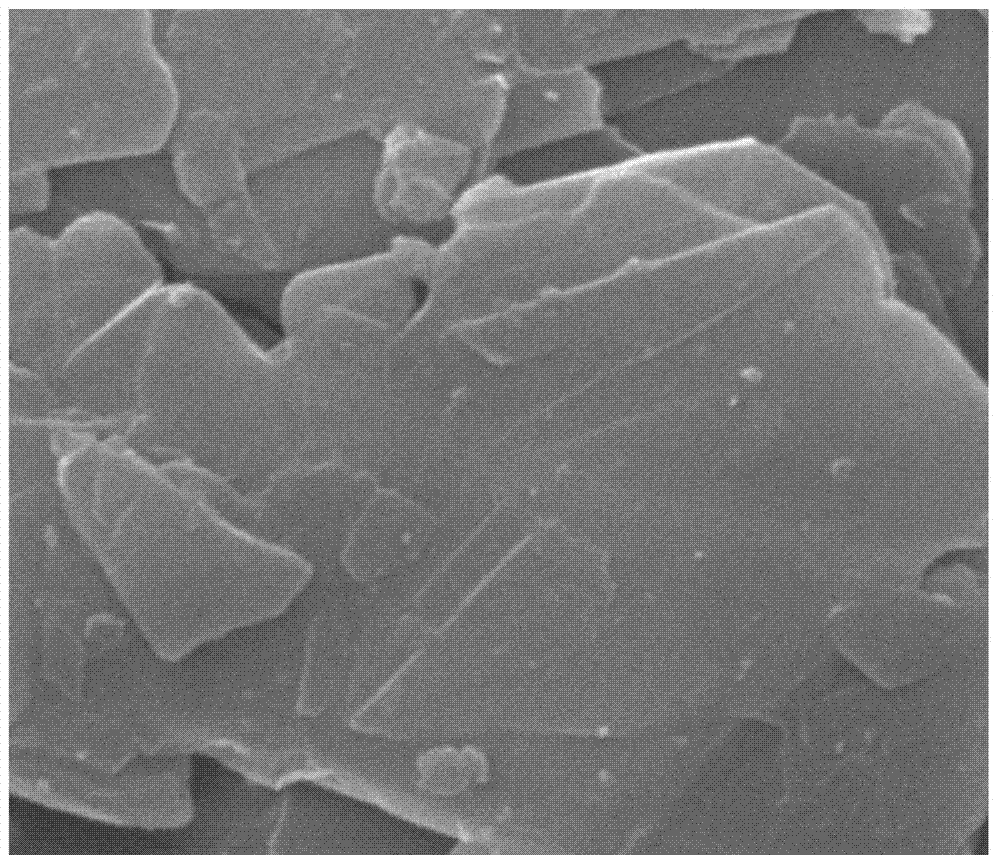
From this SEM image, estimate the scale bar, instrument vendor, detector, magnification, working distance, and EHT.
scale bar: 2000 nm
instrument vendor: FEI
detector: ETD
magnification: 25 K X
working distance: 12.2 mm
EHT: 20 kV